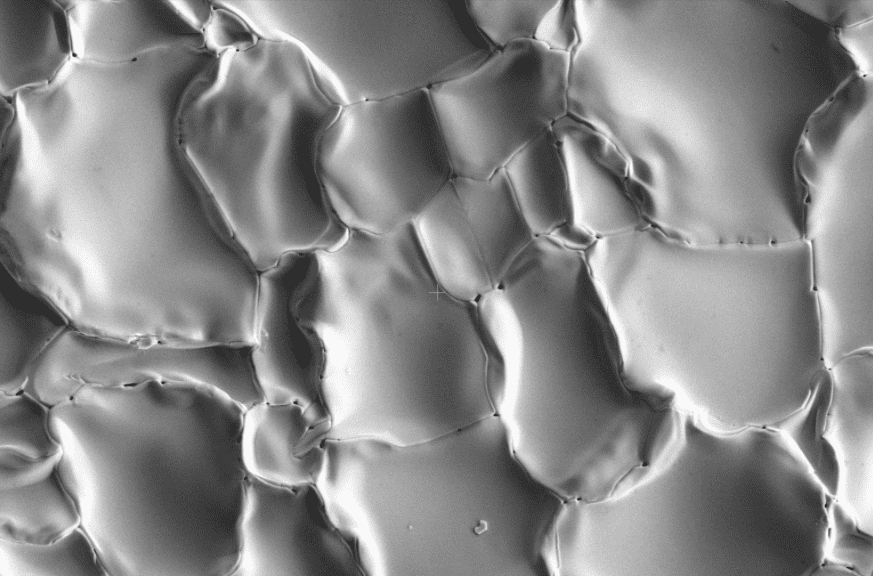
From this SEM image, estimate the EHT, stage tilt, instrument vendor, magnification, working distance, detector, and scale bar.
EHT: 10 kV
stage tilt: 0°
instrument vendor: FEI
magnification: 2 K X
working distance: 11.3 mm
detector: ETD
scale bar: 10000 nm